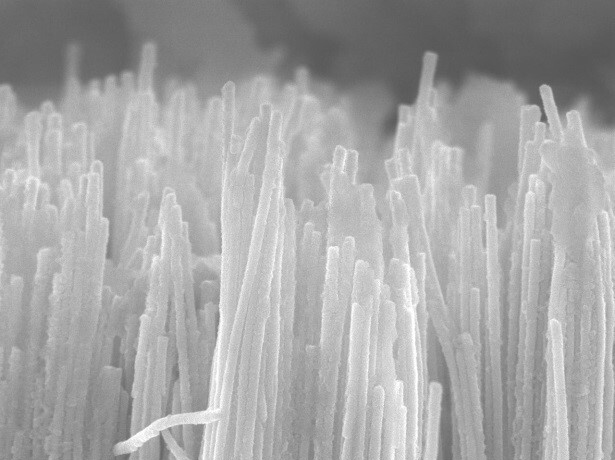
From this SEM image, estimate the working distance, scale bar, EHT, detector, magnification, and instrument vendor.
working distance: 8 mm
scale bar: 1000 nm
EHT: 15 kV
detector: SEI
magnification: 20 K X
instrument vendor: JEOL